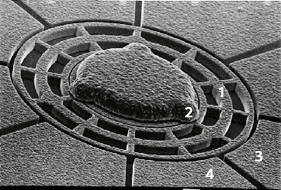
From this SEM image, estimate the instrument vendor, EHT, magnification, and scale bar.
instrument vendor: JEOL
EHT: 30 kV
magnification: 0.75 K X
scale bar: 10000 nm